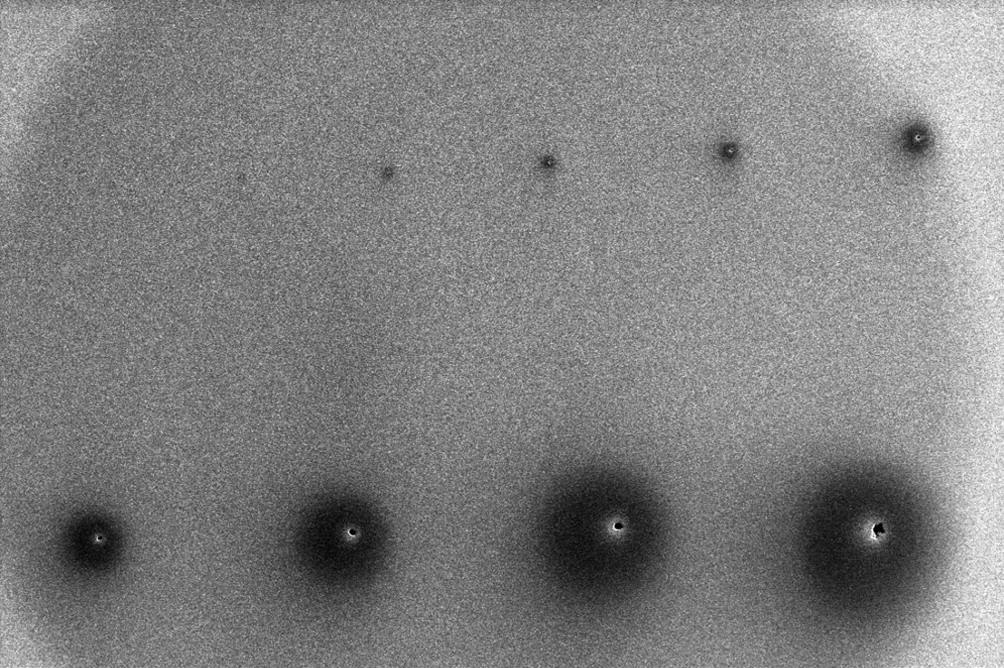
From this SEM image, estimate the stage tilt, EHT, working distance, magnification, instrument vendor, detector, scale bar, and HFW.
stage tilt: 0°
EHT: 5 kV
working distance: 4.1 mm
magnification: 0.772 K X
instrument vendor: FEI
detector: ETD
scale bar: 200000 nm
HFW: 537 µm